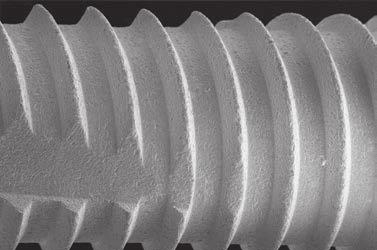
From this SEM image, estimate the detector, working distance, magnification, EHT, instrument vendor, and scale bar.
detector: SE1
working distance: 16 mm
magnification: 0.062 K X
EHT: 18 kV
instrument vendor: Zeiss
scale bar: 300000 nm